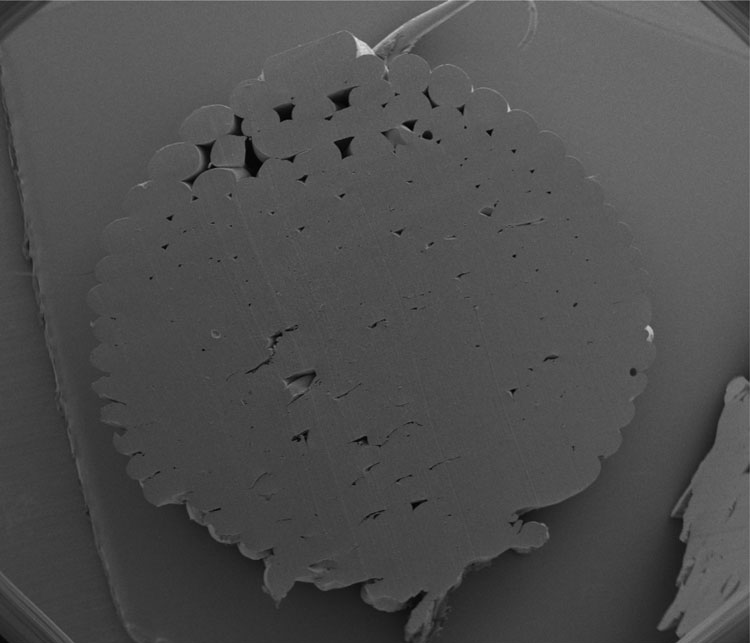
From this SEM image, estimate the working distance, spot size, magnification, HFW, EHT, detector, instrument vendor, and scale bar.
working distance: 7.5 mm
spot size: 2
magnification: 0.05 K X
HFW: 5970 µm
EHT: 5 kV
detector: ETD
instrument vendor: FEI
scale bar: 1000000 nm